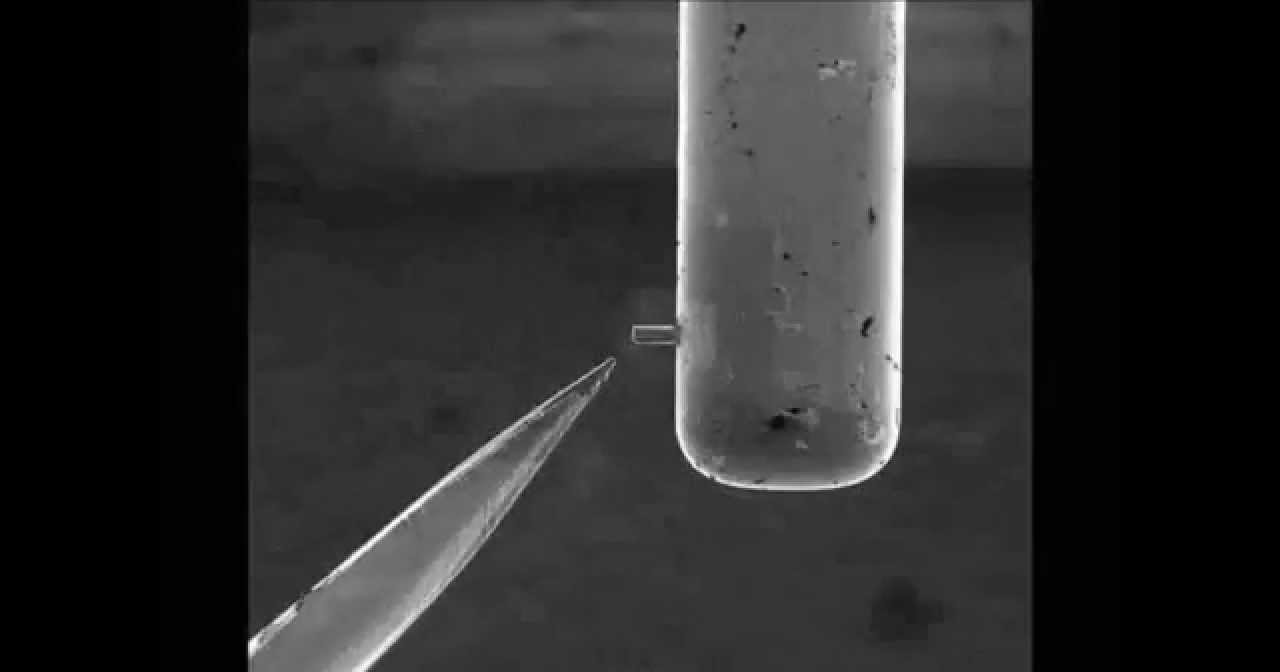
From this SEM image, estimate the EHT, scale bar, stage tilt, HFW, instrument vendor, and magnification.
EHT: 30 kV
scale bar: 50000 nm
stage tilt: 0°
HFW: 197 µm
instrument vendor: FEI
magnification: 0.65 K X